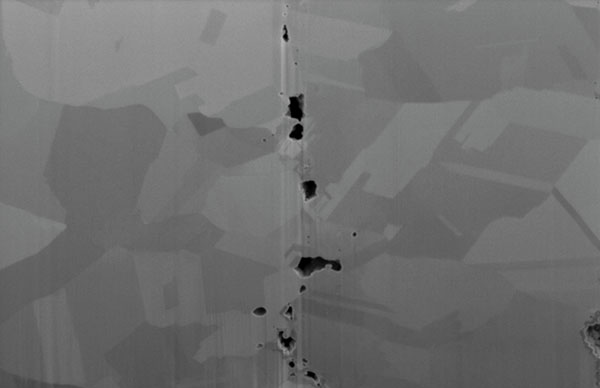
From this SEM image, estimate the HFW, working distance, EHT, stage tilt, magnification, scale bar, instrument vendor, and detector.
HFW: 8.29 µm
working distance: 4 mm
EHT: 2 kV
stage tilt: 52°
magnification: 25 K X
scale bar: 3000 nm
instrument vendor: FEI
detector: SE